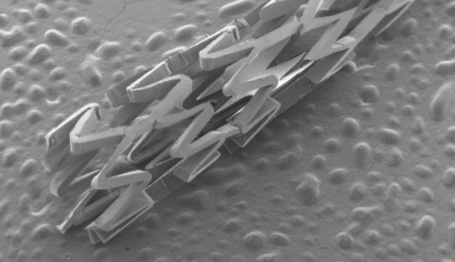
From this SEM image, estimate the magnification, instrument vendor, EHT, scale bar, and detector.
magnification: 0.02 K X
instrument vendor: JEOL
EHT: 15 kV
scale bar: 1000000 nm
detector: SEI